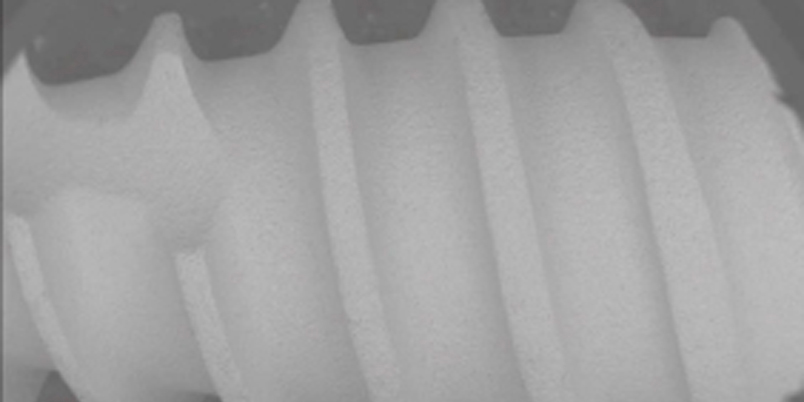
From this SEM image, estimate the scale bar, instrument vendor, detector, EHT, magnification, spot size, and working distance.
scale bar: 3000000 nm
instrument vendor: FEI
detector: BSED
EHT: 20 kV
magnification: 0.05 K X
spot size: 4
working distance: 10.3 mm